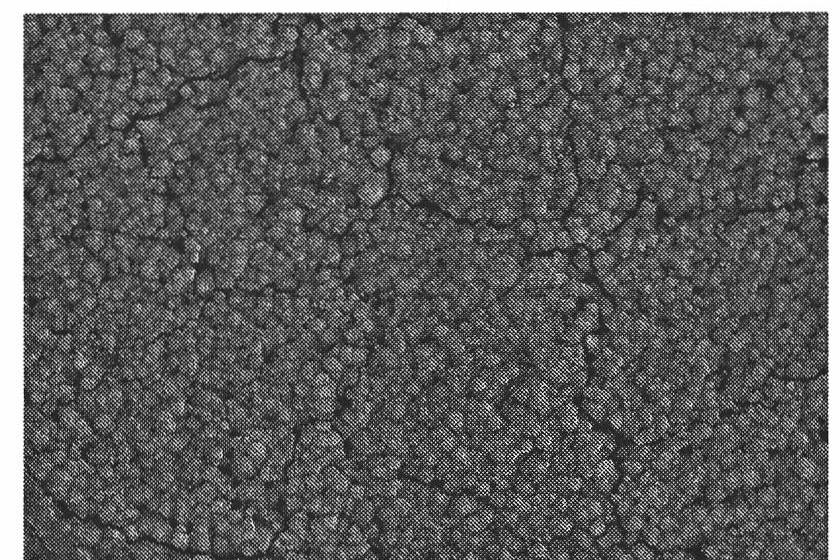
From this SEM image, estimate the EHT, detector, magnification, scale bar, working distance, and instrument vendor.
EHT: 15 kV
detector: SE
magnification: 10 K X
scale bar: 5000 nm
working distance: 6.4 mm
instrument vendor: Hitachi